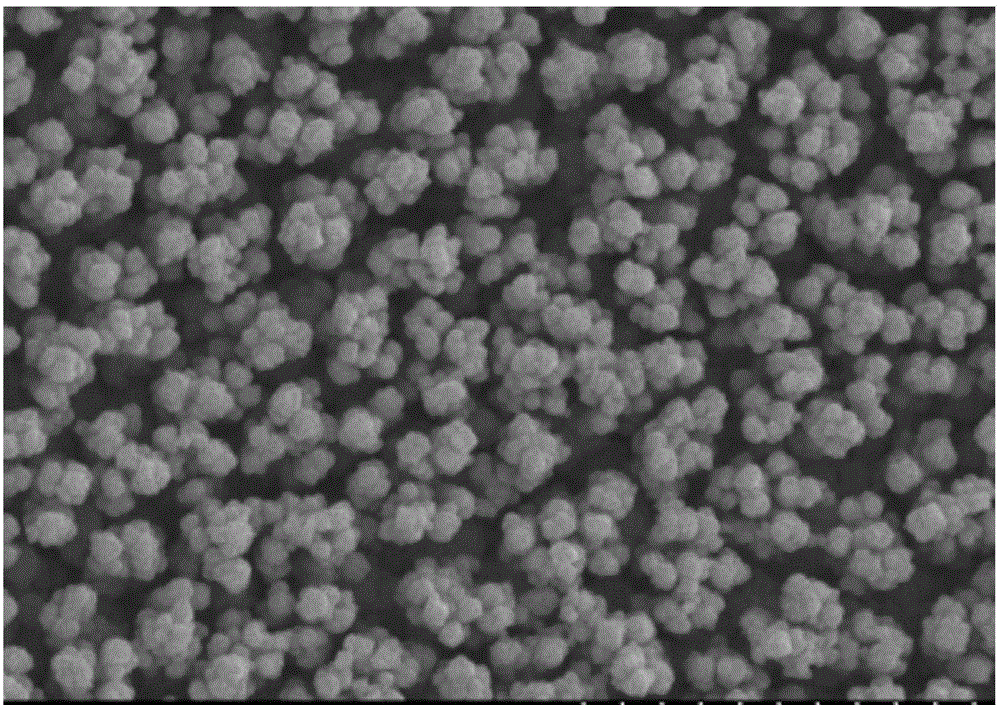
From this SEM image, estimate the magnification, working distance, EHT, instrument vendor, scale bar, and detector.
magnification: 5 K X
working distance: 12 mm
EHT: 15 kV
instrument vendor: Hitachi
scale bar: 10000 nm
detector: SE